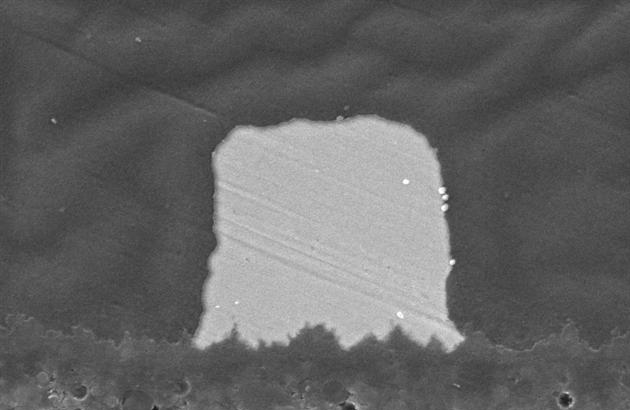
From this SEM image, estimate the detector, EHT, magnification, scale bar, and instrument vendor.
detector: SEI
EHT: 15 kV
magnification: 3 K X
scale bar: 5000 nm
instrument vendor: JEOL